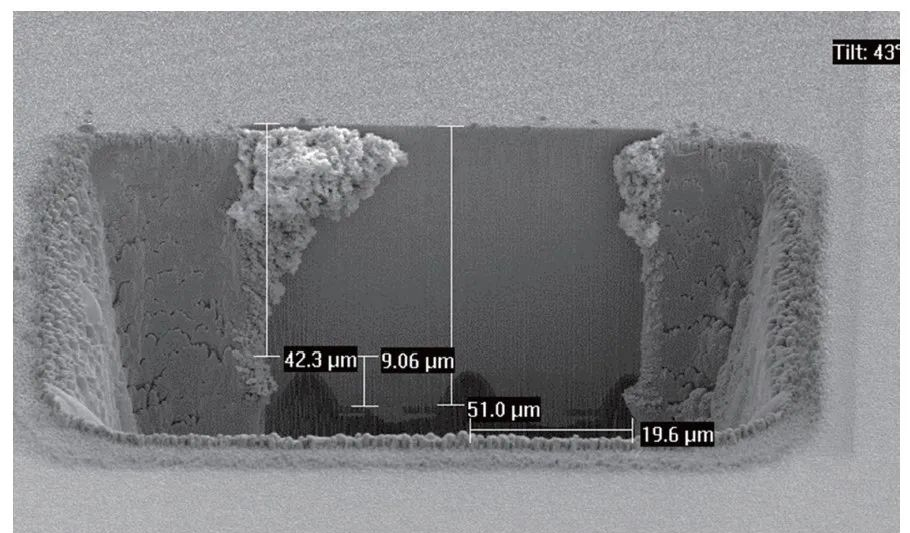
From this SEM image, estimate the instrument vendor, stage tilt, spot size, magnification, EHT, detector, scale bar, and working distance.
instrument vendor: FEI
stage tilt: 43°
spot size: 3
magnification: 1.16 K X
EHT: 5 kV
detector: SE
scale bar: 20000 nm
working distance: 5.2 mm